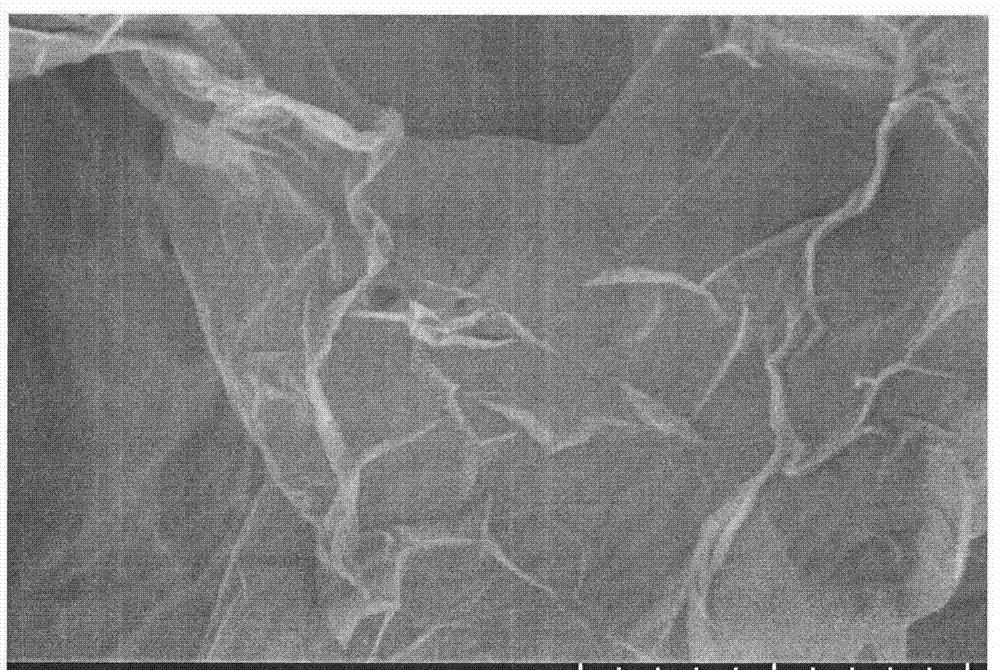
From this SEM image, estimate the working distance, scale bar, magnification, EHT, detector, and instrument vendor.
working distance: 4.6 mm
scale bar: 1000 nm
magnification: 50 K X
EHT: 1 kV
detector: SE+BSE(U)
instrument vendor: Hitachi